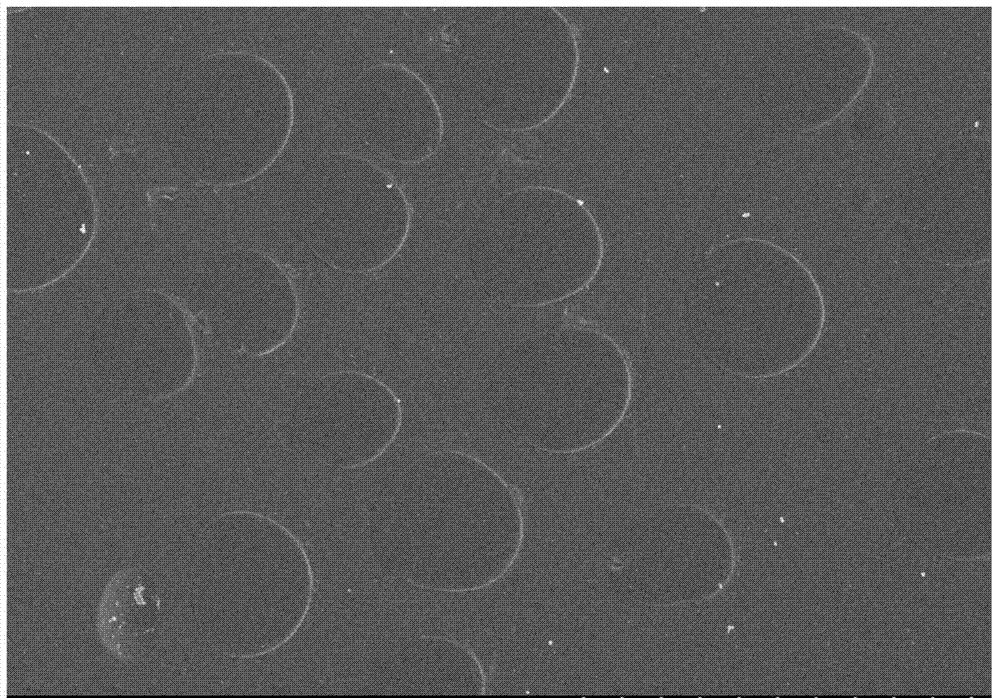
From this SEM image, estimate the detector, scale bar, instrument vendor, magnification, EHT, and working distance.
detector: SE(M)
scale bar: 10000 nm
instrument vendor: Hitachi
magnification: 5 K X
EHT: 3 kV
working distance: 9.9 mm